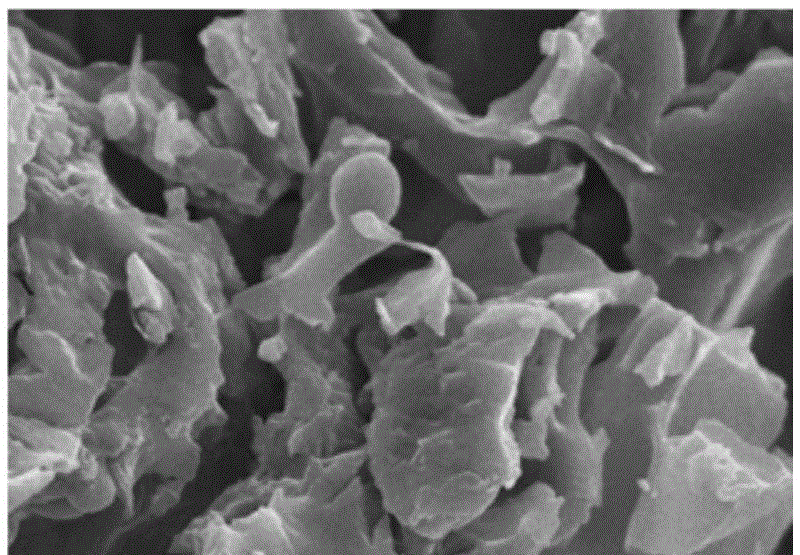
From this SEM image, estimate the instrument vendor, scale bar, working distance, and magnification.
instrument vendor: Hitachi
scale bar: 10000 nm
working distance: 10.6 mm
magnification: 3 K X